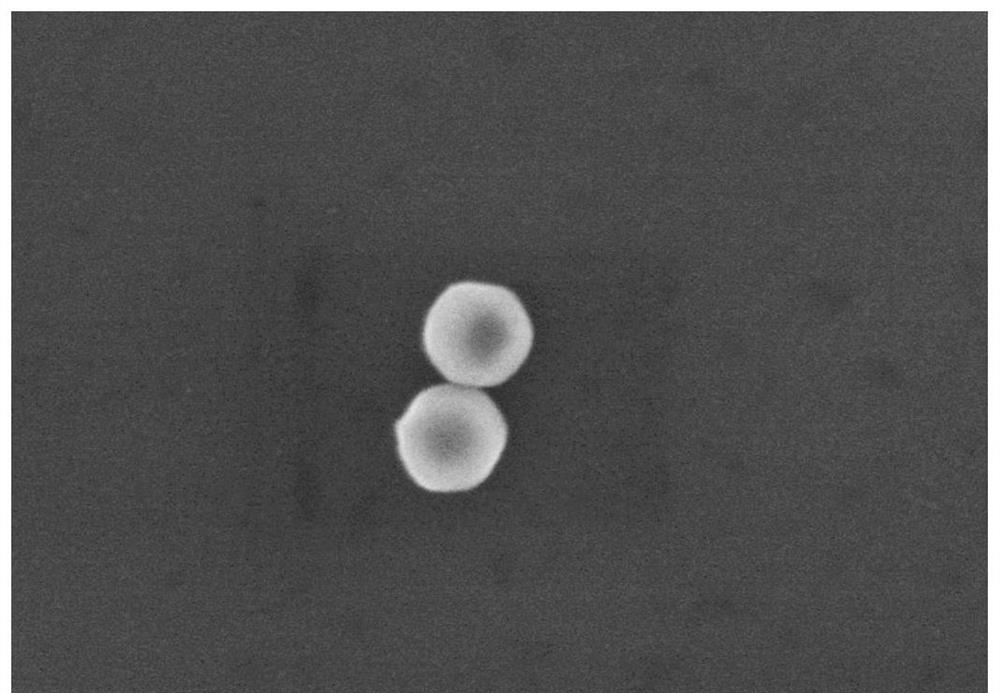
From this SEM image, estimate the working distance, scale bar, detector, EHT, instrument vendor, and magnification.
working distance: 10.1 mm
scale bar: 500 nm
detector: SE(M)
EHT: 5 kV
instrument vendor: Hitachi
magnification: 80 K X